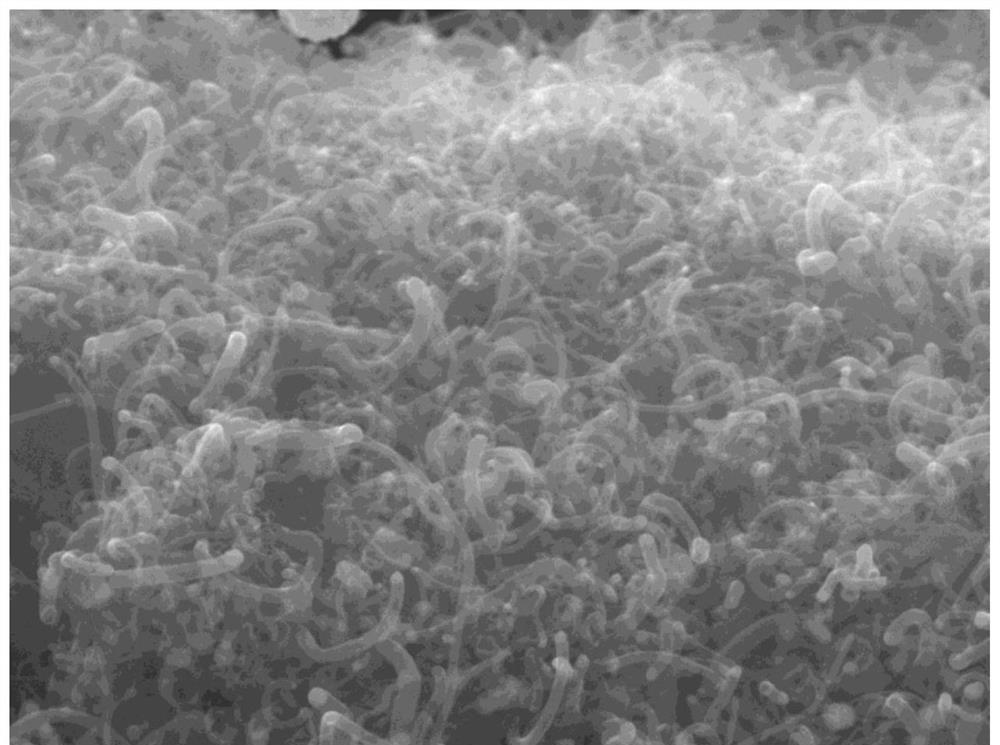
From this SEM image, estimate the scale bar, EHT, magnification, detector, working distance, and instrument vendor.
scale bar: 100 nm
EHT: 15 kV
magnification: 30 K X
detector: SEI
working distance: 9.9 mm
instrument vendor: JEOL